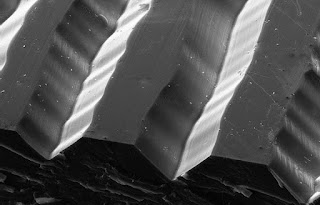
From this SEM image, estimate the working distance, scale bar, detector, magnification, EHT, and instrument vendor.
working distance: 11.1 mm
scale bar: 10000 nm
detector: SE2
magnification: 0.34 K X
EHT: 20 kV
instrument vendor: Zeiss